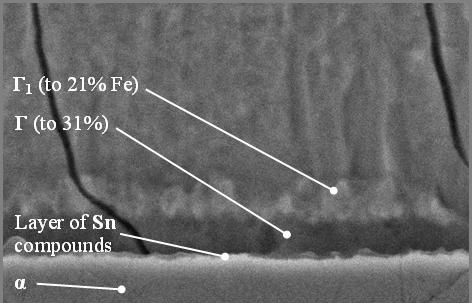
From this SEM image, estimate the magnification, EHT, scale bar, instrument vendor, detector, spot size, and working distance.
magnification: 5 K X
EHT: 20 kV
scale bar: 5000 nm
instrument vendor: FEI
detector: SE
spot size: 2.6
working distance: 6.6 mm